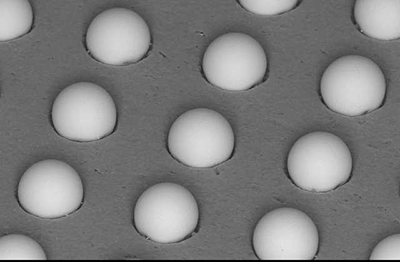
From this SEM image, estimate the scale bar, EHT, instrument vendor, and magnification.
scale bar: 50000 nm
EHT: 15 kV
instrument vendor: other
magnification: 0.5 K X